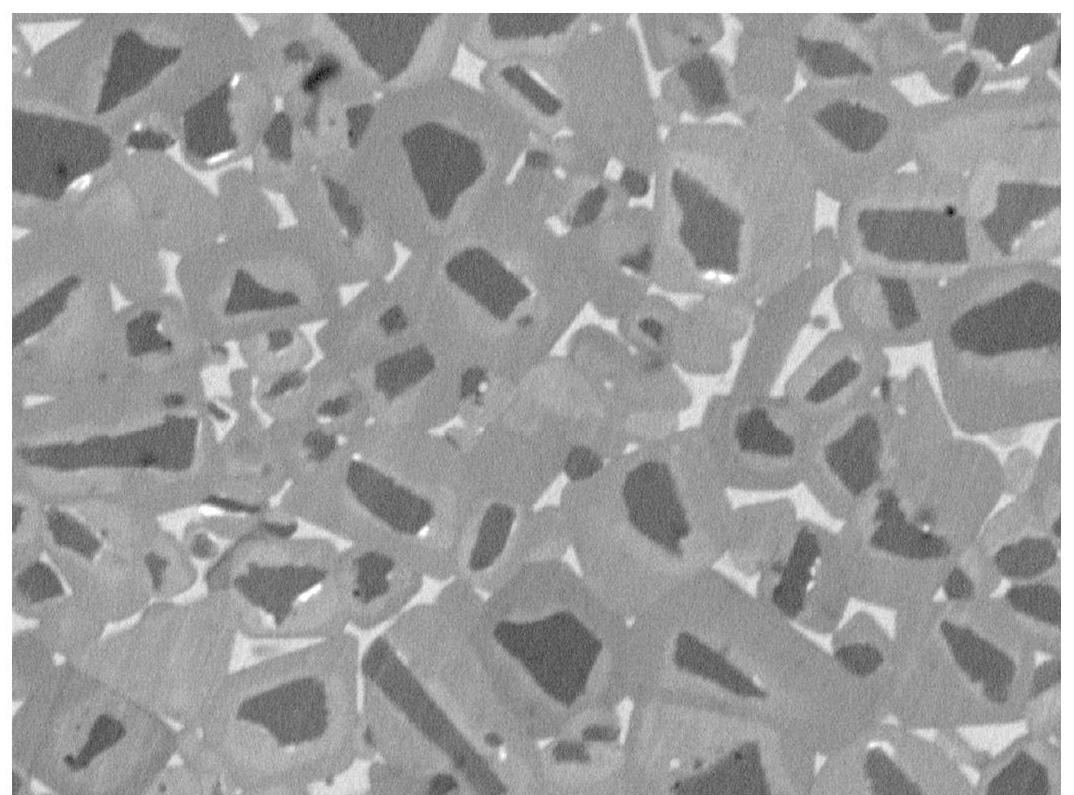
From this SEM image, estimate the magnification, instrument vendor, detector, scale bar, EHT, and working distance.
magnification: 7 K X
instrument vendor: JEOL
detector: BED-C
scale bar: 1000 nm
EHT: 5 kV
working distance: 8.6 mm